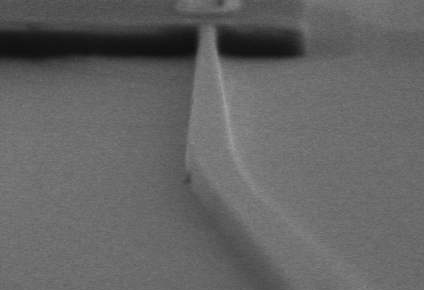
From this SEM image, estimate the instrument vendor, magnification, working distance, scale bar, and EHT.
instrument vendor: Hitachi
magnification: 3 K X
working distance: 10.8 mm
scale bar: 10000 nm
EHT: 10 kV